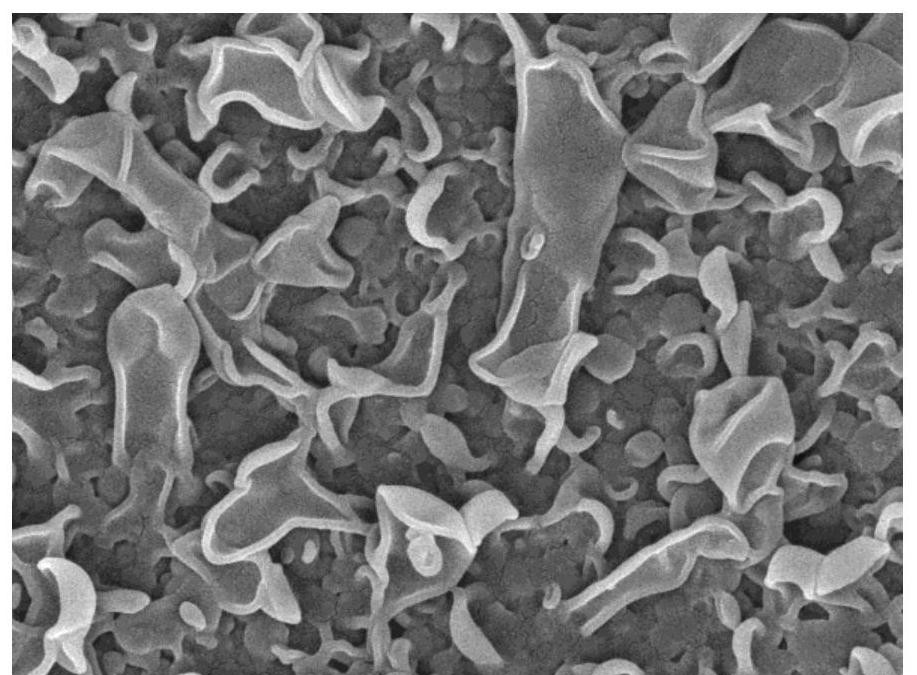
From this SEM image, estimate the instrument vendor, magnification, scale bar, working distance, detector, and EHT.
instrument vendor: JEOL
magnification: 50 K X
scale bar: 100 nm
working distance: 4.3 mm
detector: SEI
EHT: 5 kV